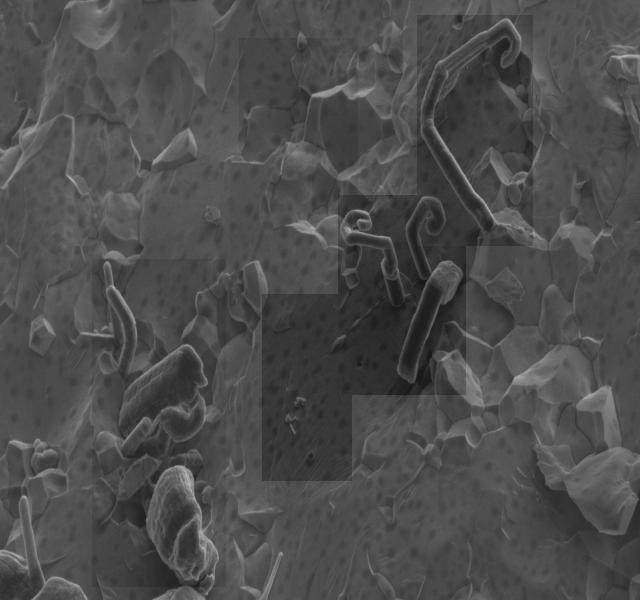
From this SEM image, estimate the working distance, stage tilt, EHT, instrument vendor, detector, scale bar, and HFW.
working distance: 4.5 mm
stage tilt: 0°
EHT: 2 kV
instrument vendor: FEI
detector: ETD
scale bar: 10000 nm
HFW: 27.6 µm